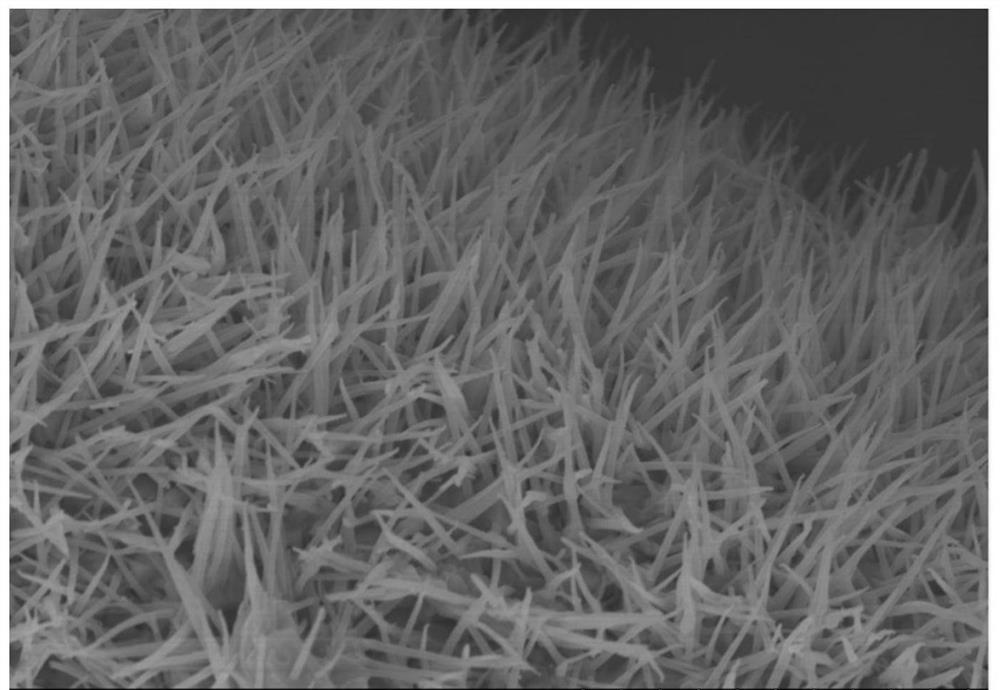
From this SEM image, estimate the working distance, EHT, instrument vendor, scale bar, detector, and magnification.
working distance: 10.9 mm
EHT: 10 kV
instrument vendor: Hitachi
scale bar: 5000 nm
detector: SE(U)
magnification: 10 K X